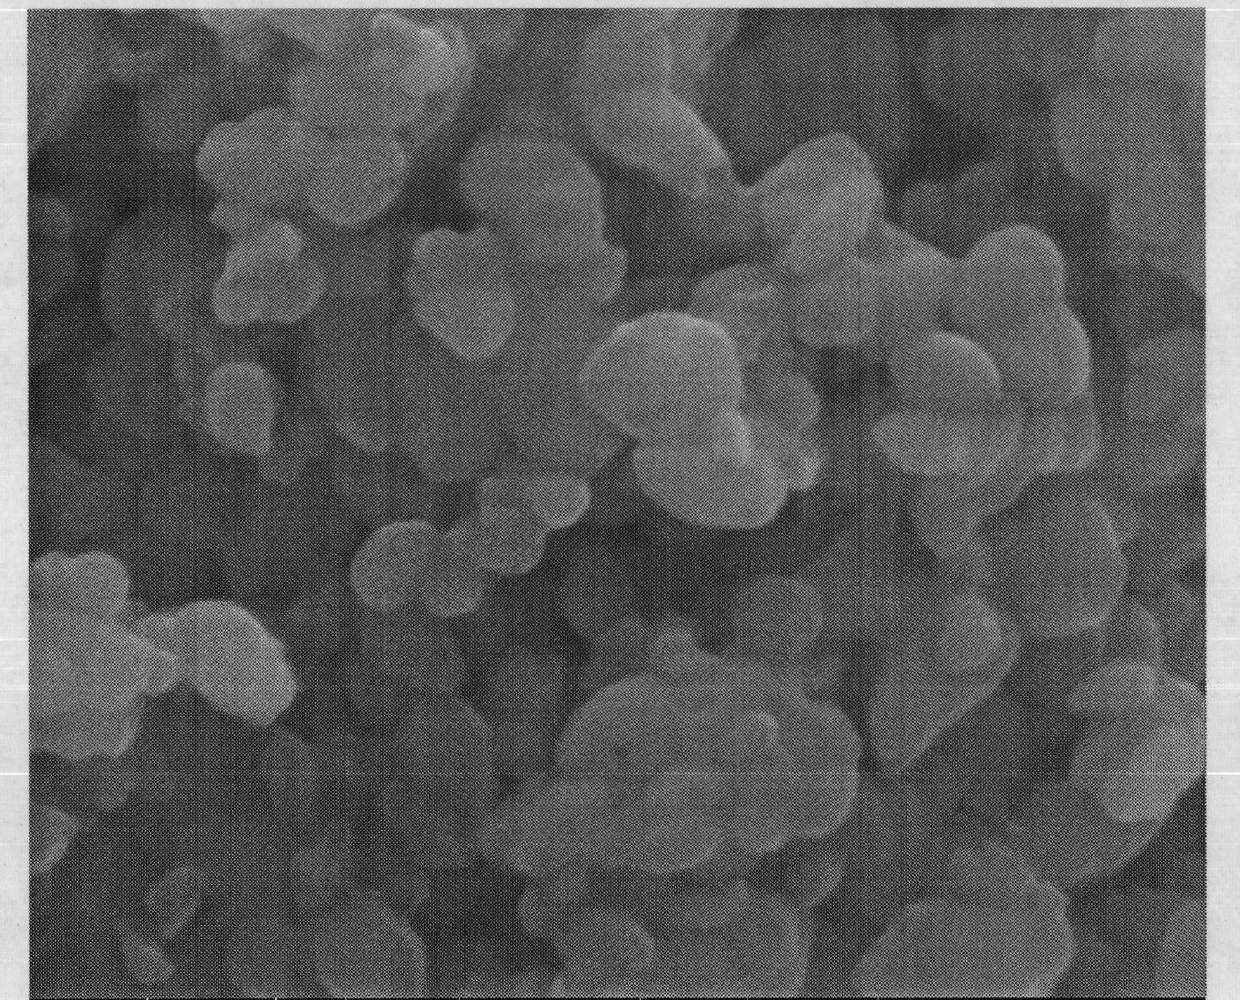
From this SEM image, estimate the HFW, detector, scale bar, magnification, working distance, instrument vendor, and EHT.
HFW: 2.7 µm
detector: ETD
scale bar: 500 nm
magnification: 50 K X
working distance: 11.2 mm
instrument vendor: FEI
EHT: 20 kV